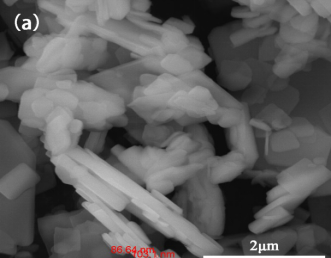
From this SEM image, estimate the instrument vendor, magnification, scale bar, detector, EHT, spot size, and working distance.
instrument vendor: FEI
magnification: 50 K X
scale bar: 2000 nm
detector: ETD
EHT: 20 kV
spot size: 3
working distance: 10.9 mm